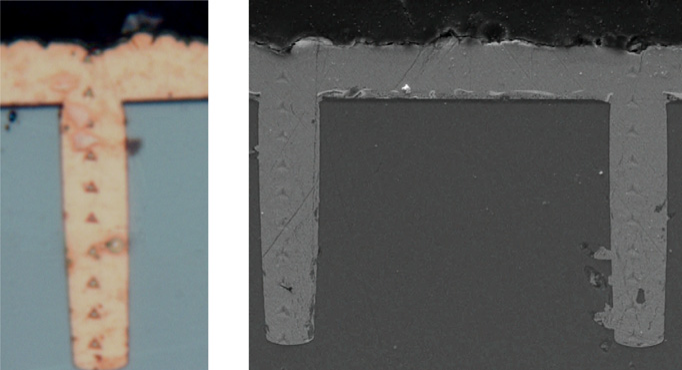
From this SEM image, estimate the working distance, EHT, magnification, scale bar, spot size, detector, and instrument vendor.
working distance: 6.8 mm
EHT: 5 kV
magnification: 8 K X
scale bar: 10000 nm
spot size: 3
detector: ETD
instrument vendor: FEI